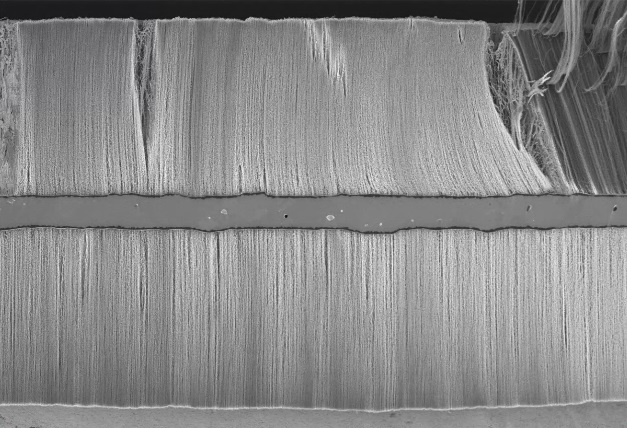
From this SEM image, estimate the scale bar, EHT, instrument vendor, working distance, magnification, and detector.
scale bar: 100000 nm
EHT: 2 kV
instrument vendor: Hitachi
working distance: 11.7 mm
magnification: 0.45 K X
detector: SE(U)